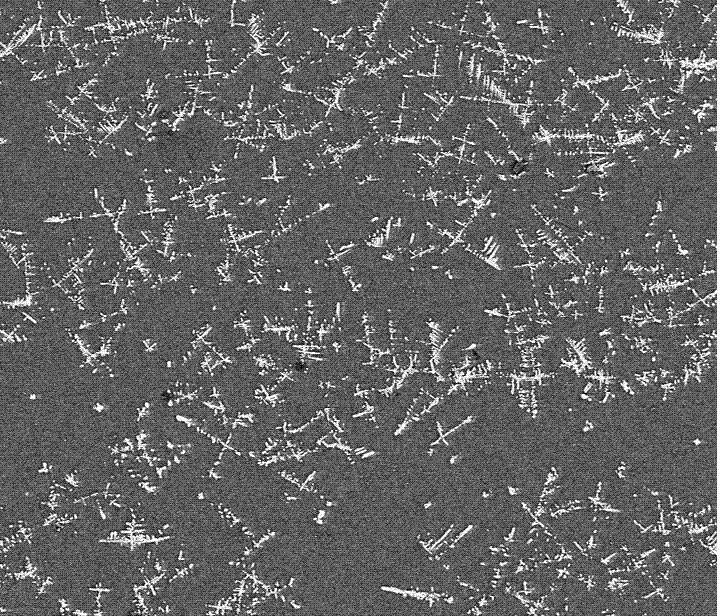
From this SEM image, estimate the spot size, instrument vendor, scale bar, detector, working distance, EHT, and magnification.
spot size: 2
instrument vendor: FEI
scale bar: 200000 nm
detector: BSED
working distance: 11 mm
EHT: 20 kV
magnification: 0.2 K X